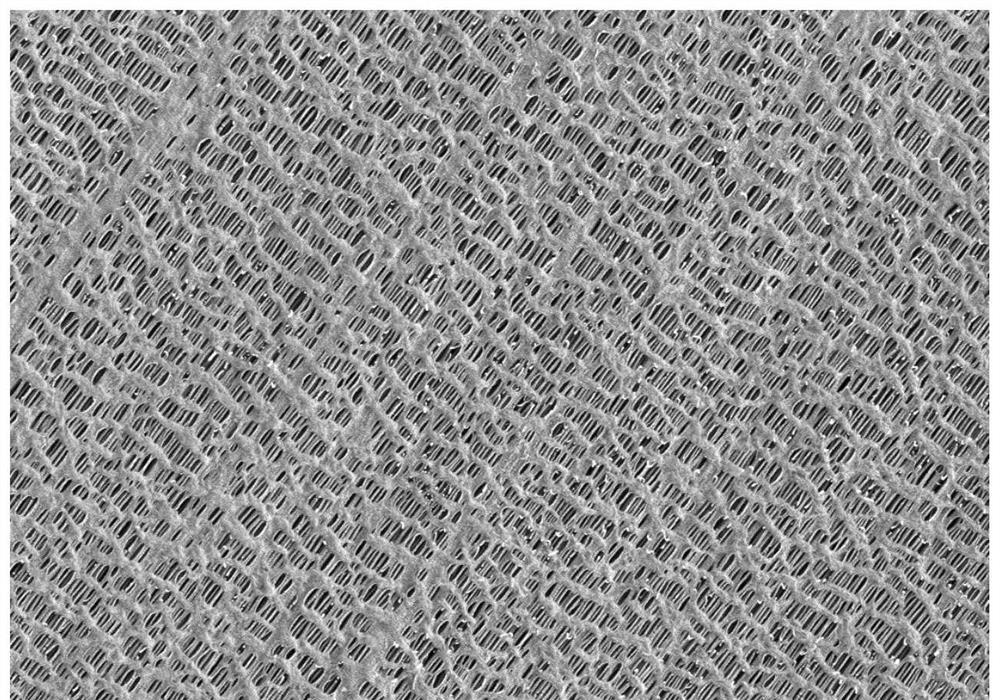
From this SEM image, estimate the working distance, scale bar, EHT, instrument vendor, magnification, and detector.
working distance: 5.4 mm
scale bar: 1000 nm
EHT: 2 kV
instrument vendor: Zeiss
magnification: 10 K X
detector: SE2